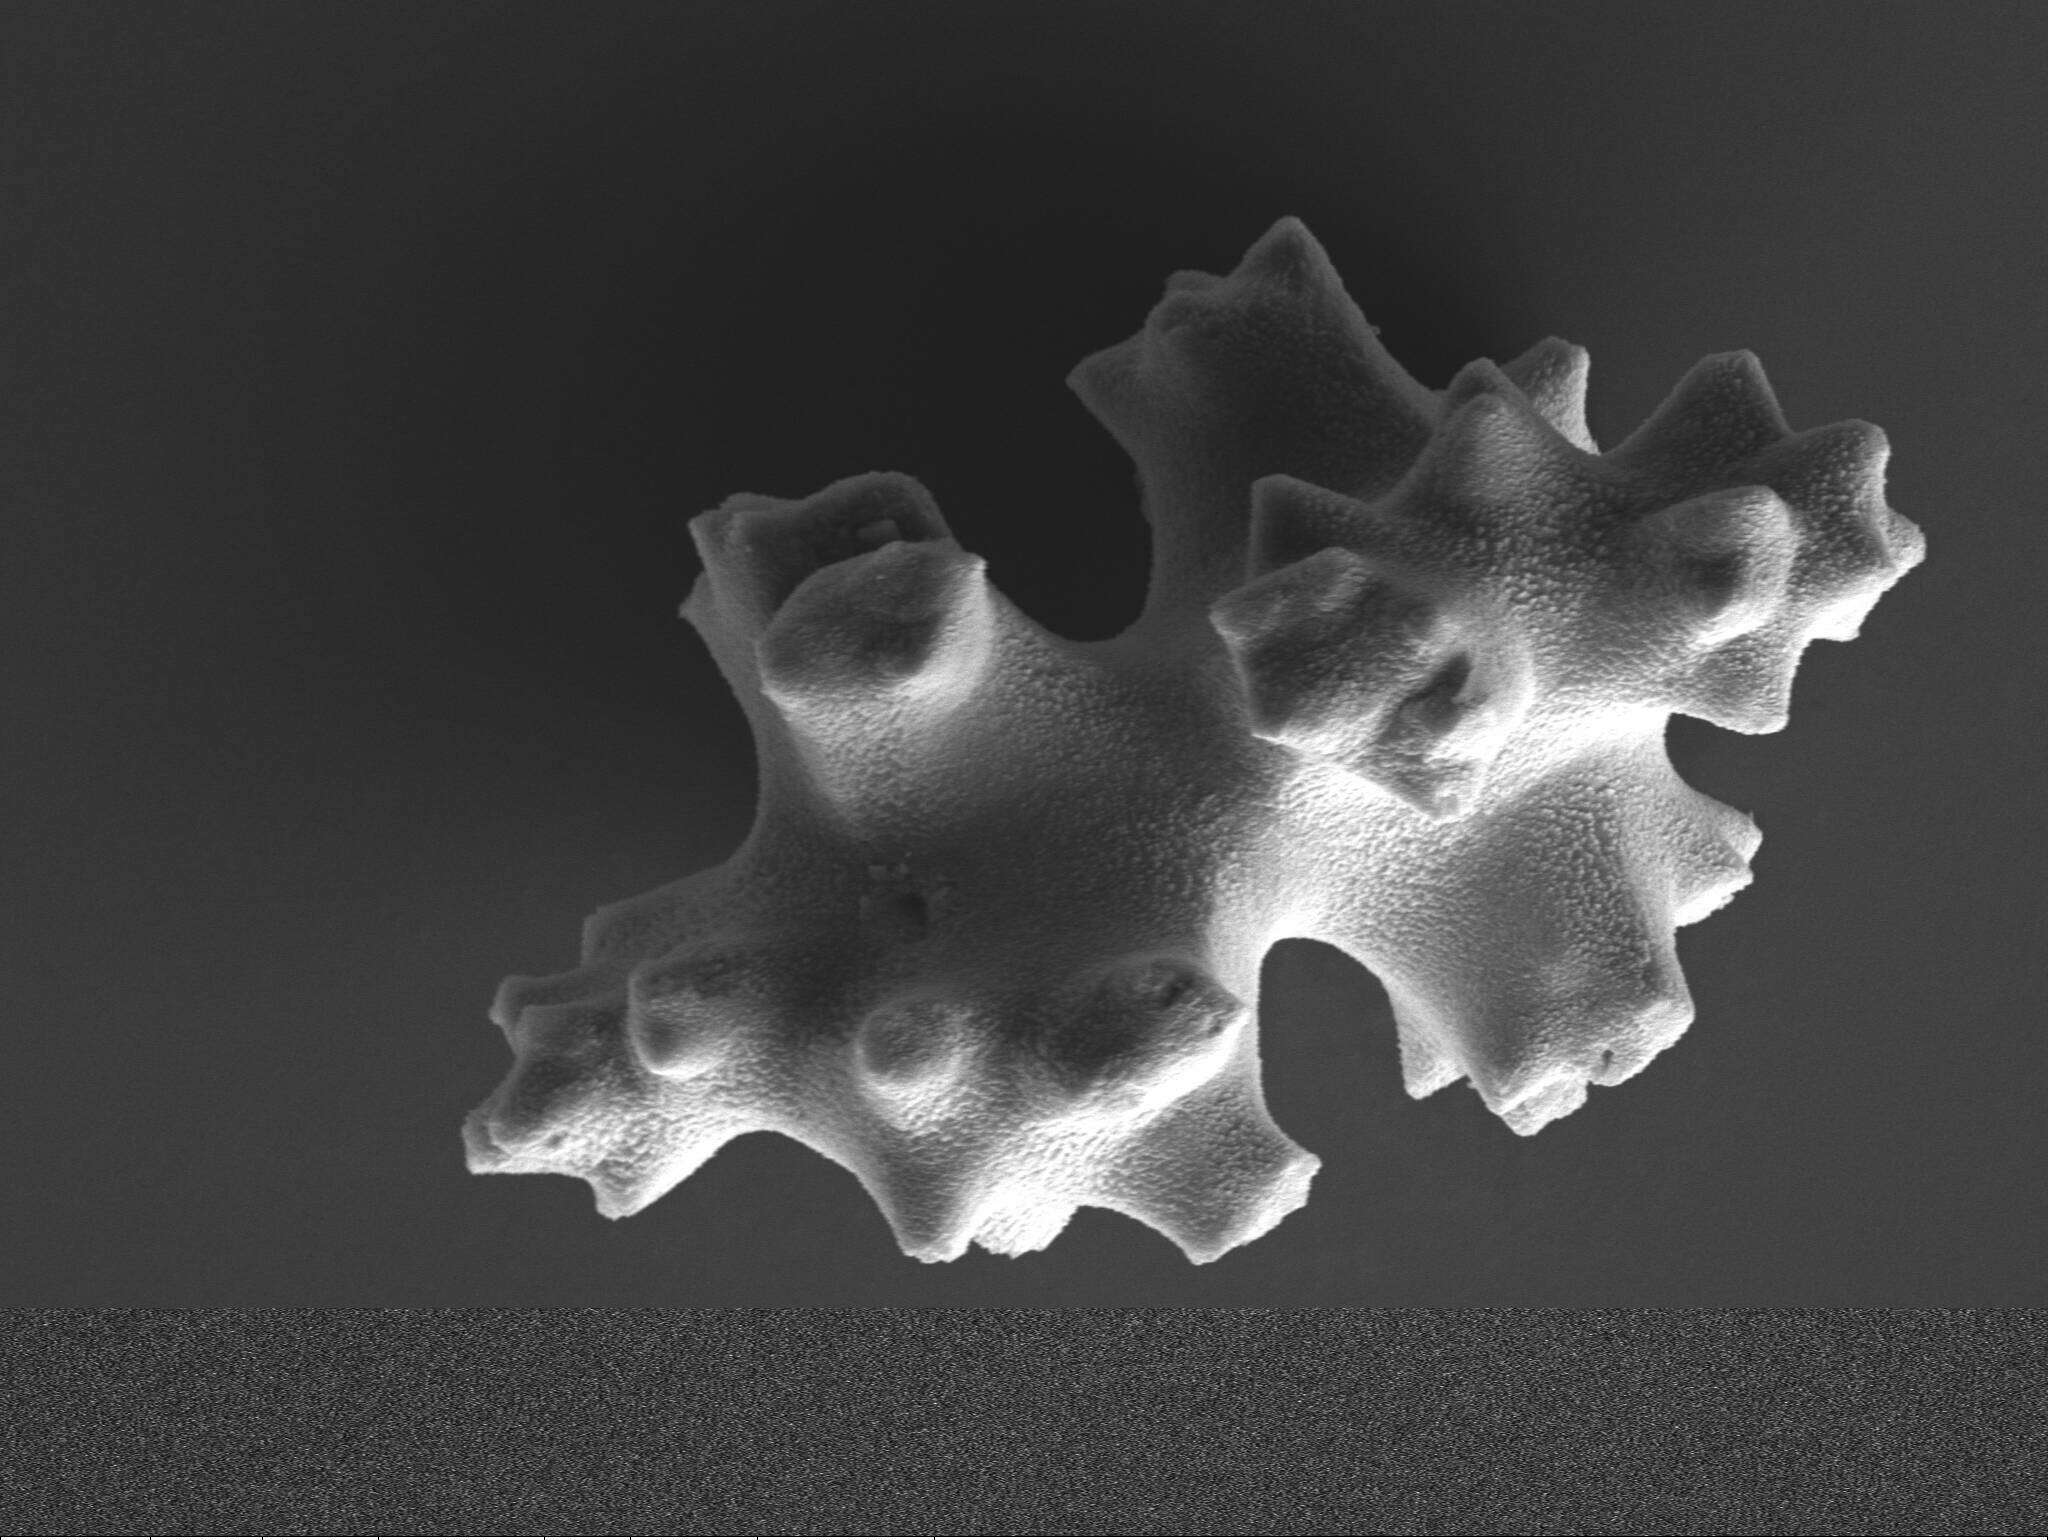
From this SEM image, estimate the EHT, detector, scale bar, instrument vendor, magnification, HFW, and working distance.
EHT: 12 kV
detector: SE1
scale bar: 20000 nm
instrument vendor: Zeiss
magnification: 1 K X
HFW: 114.4 µm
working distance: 13.3 mm